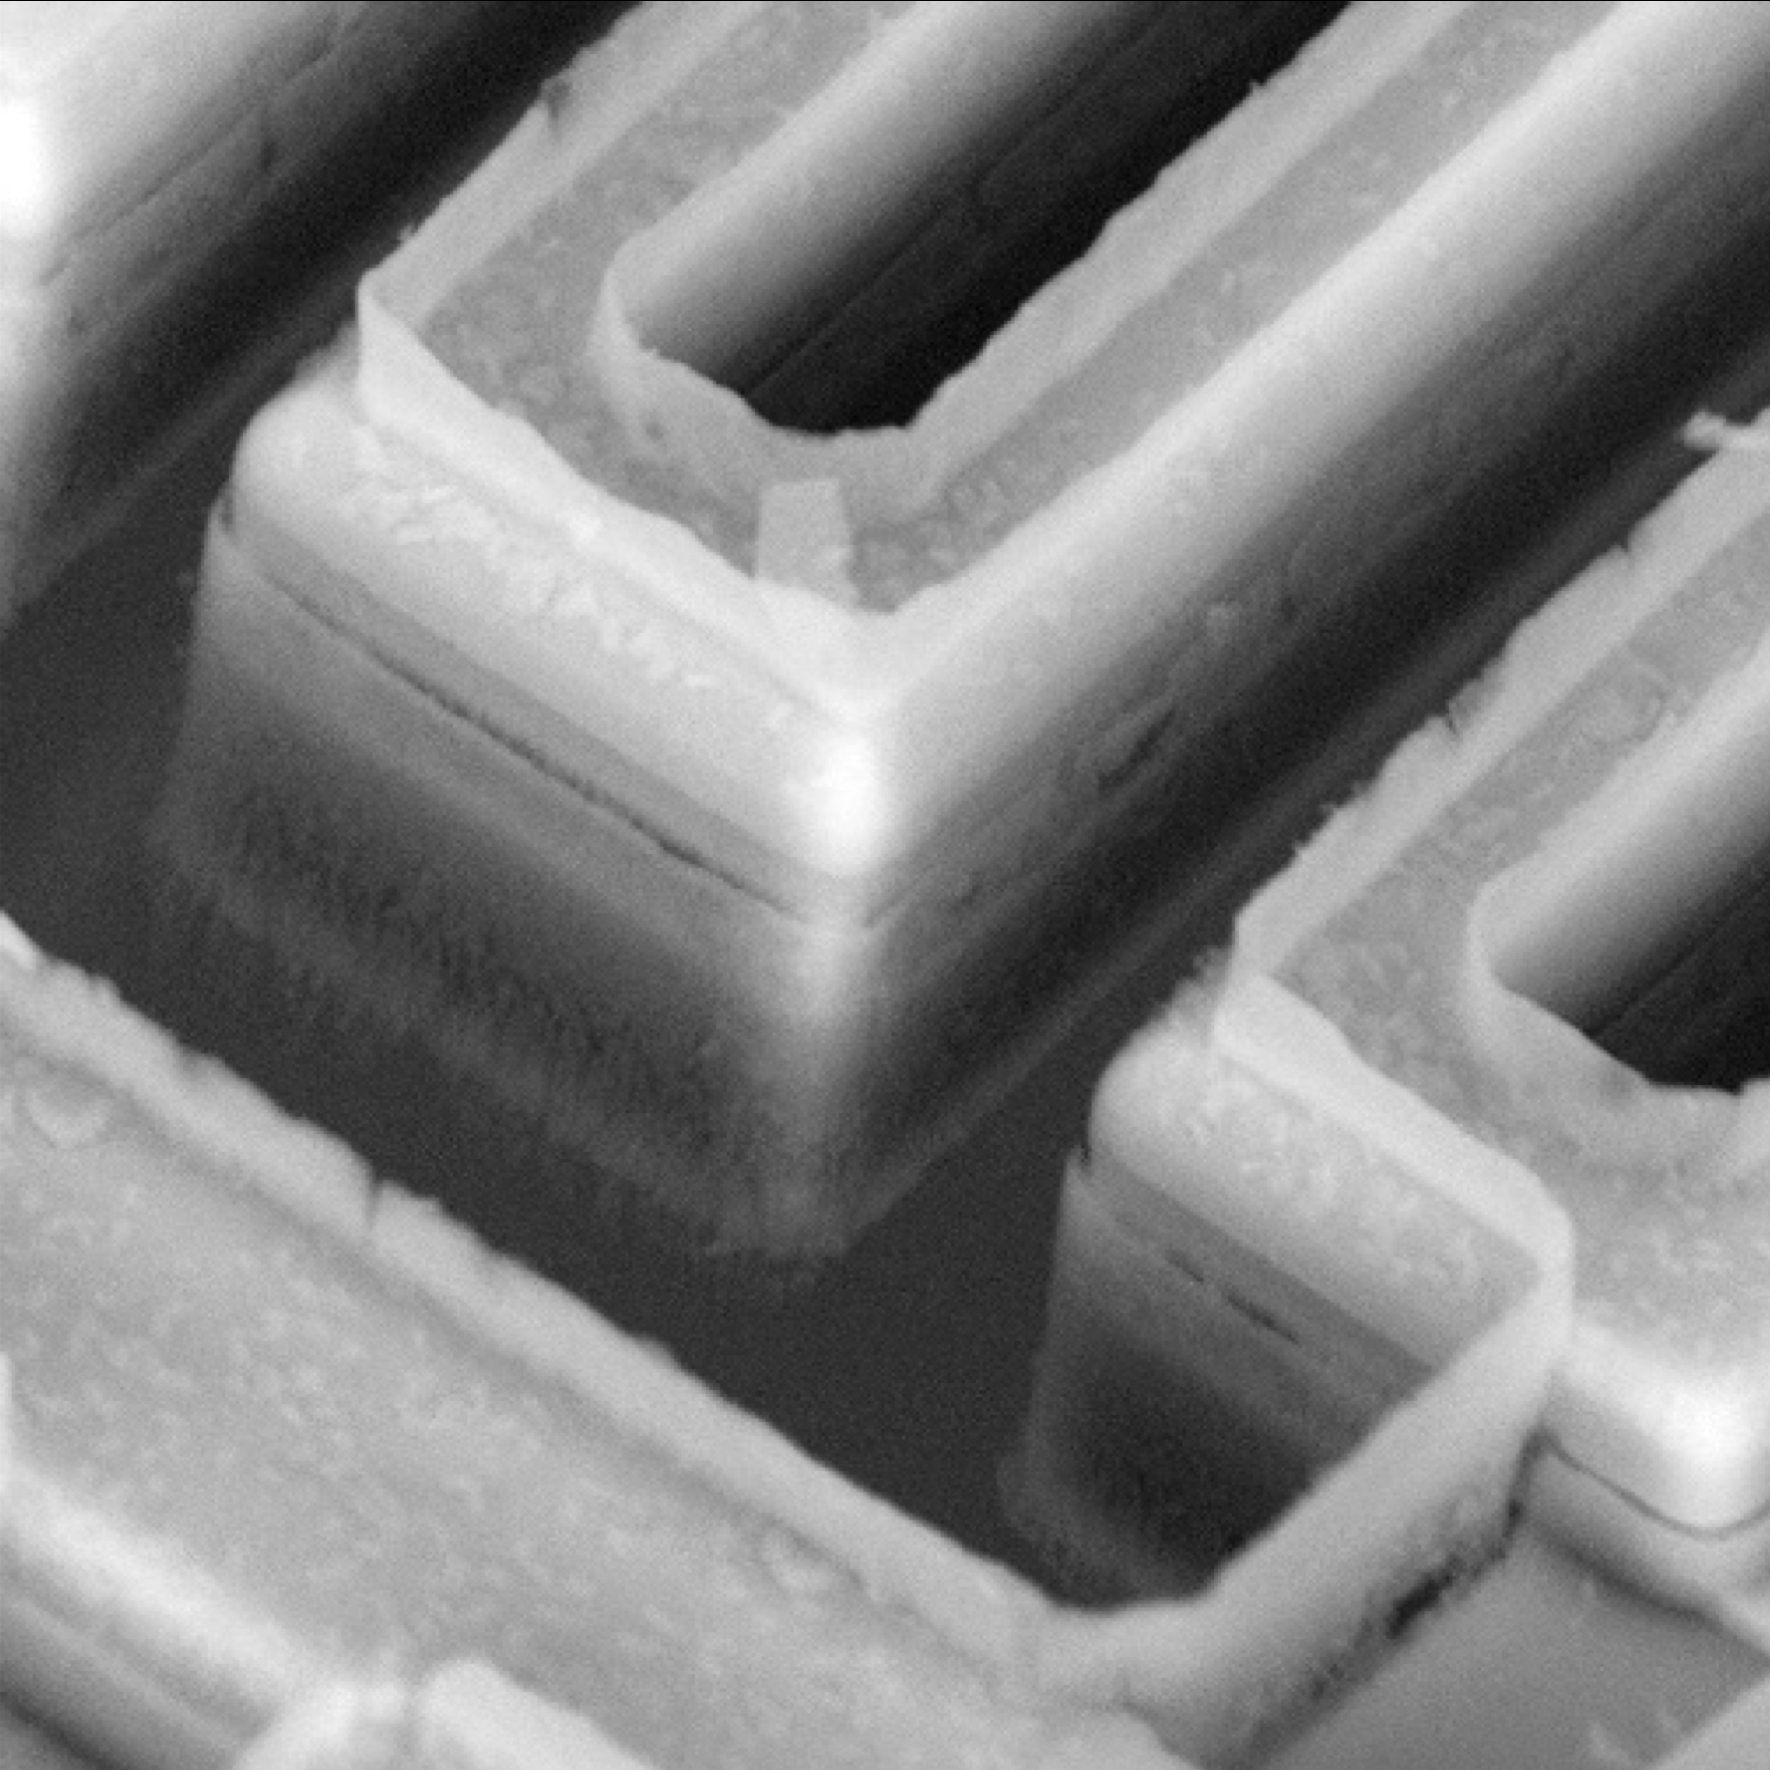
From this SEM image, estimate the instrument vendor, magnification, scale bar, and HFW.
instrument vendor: other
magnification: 10 K X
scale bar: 10000 nm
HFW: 26.8 µm